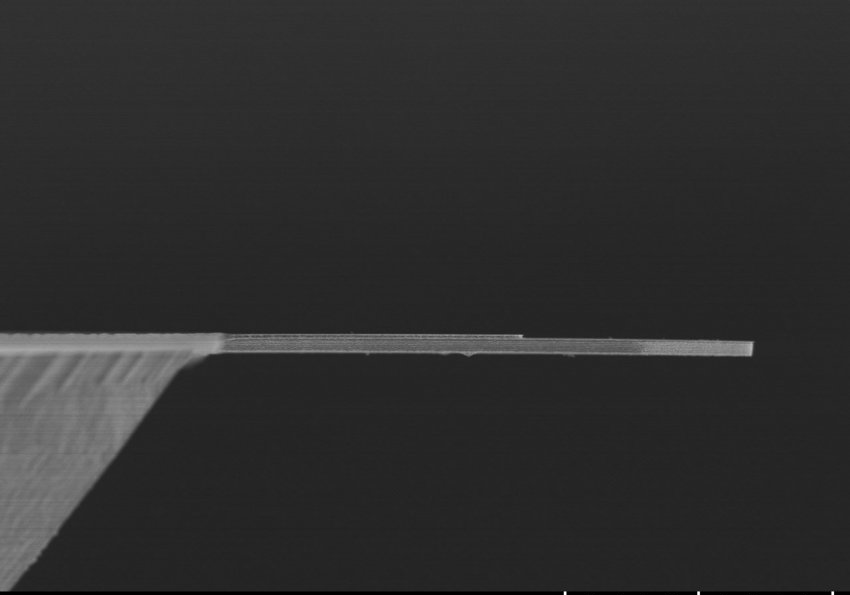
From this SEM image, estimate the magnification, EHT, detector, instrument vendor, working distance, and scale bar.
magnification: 0.8 K X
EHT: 4 kV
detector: SE(UL)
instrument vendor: Hitachi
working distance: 10.7 mm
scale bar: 50000 nm